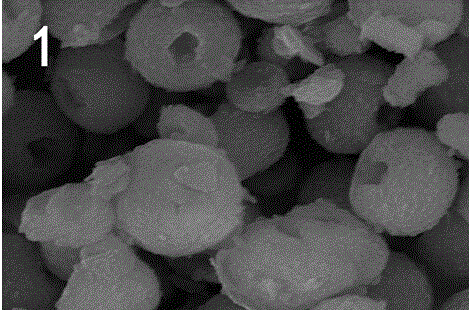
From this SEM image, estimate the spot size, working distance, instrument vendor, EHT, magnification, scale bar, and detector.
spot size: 3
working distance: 6.6 mm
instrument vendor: FEI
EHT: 15 kV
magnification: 10 K X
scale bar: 2000 nm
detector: SE